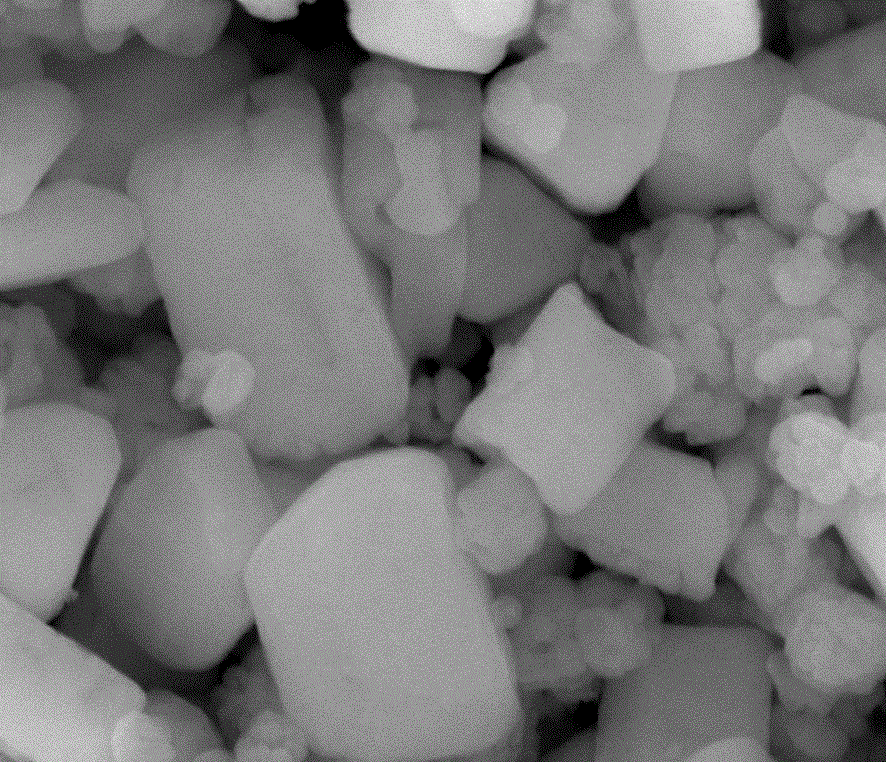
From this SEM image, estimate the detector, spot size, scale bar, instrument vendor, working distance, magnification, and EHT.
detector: ETD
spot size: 3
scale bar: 500 nm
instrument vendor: FEI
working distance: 10.3 mm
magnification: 120 K X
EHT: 20 kV